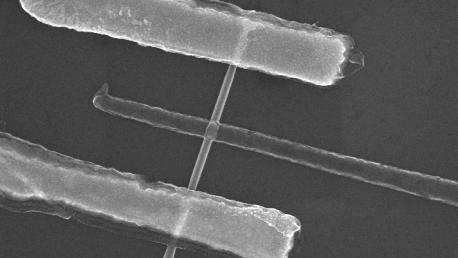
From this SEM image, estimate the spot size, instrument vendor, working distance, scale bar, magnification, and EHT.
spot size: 1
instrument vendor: FEI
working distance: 4.8 mm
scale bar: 1000 nm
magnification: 38.182 K X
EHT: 20 kV